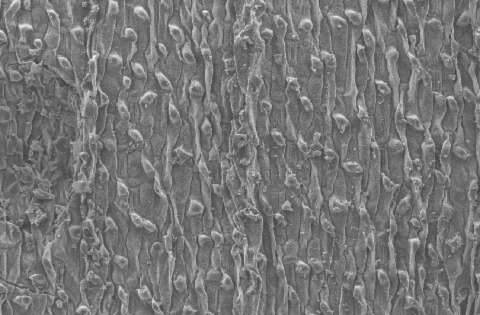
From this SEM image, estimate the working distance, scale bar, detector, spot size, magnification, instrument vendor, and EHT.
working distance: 10 mm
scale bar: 100000 nm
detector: SE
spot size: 3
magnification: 0.2 K X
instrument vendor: FEI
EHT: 10 kV